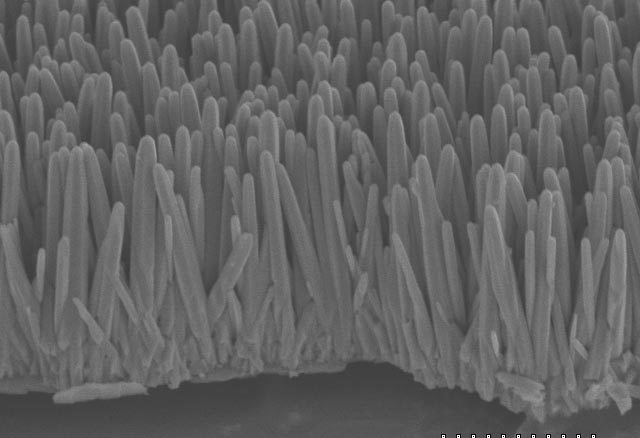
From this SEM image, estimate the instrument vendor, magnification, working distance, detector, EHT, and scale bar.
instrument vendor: Hitachi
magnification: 12 K X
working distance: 13.1 mm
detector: SE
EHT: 5 kV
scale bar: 2500 nm